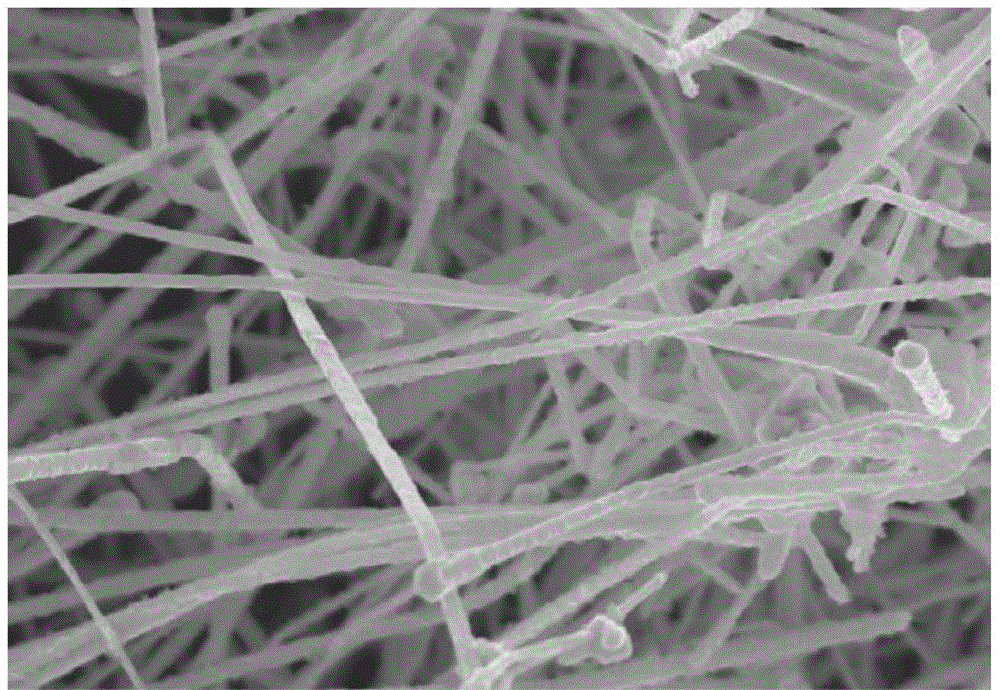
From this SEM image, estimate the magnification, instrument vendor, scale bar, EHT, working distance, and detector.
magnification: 19.51 K X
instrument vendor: Zeiss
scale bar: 200 nm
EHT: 8 kV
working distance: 3 mm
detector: InLens